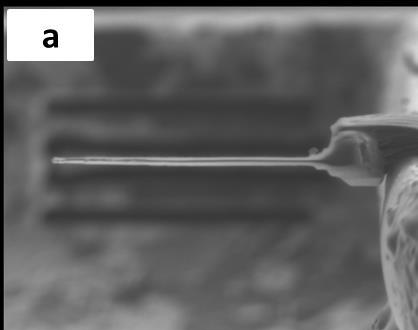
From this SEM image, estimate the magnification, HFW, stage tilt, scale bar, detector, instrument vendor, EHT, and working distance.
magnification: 7.5 K X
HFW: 17.1 µm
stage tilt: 52°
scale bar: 5000 nm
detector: ETD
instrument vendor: FEI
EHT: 30 kV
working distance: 16.4 mm